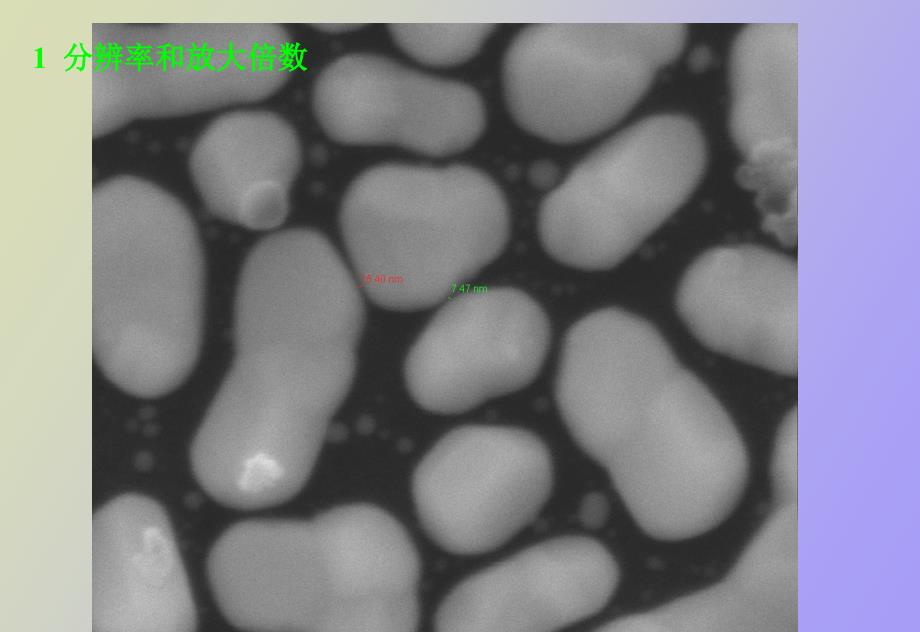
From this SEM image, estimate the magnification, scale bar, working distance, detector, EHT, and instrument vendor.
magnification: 100 K X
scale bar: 1000 nm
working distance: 7.7 mm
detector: ETD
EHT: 25 kV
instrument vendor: FEI